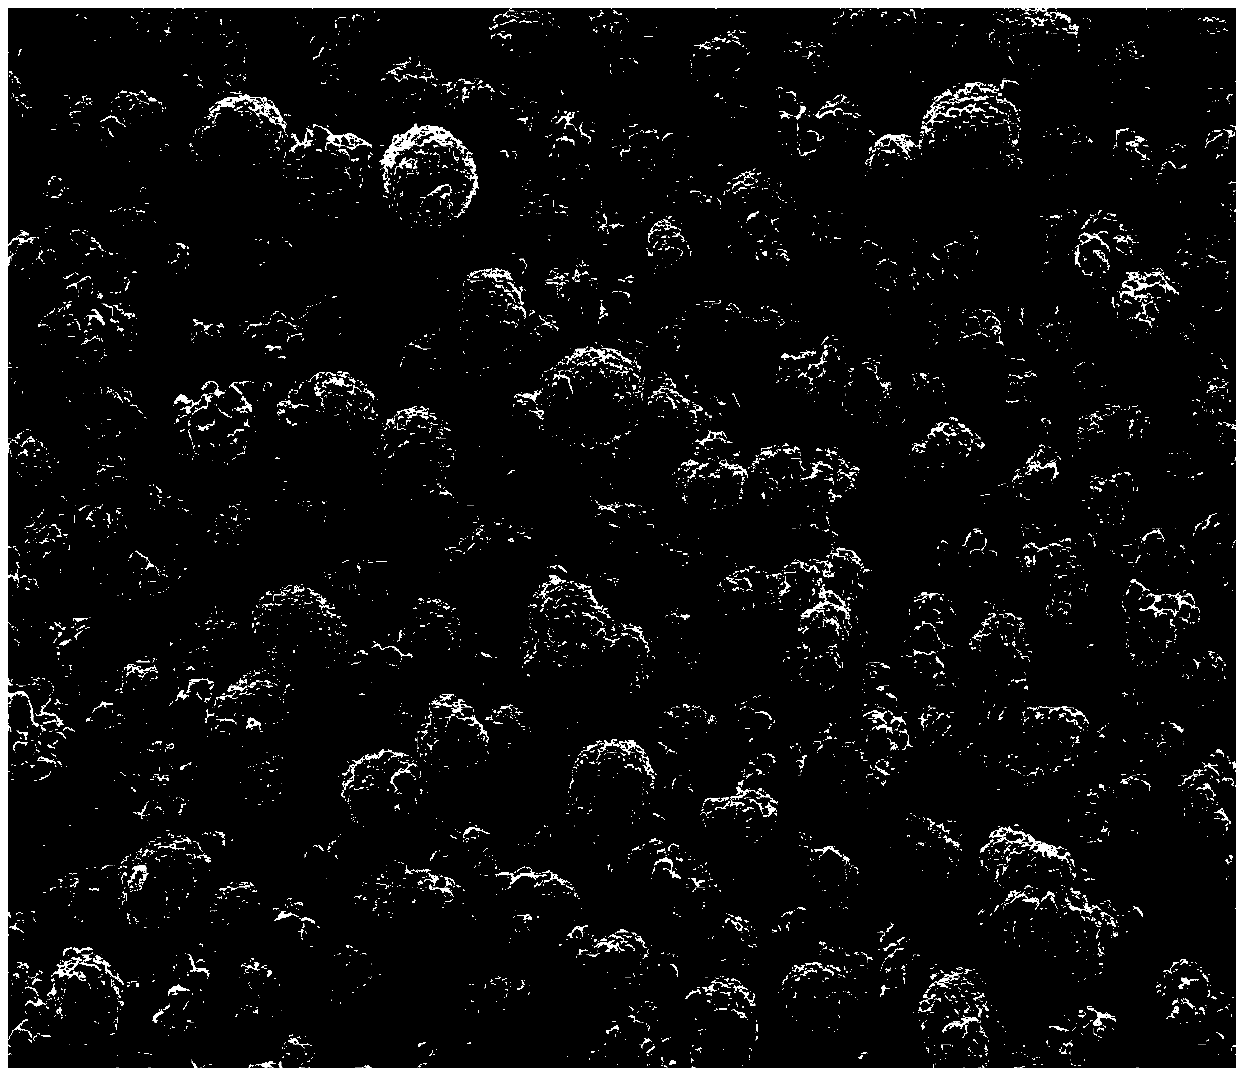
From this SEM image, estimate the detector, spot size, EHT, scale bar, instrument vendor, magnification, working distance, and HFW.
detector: ETD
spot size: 2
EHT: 10 kV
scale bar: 40000 nm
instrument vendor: FEI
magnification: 1 K X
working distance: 7.8 mm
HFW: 149 µm